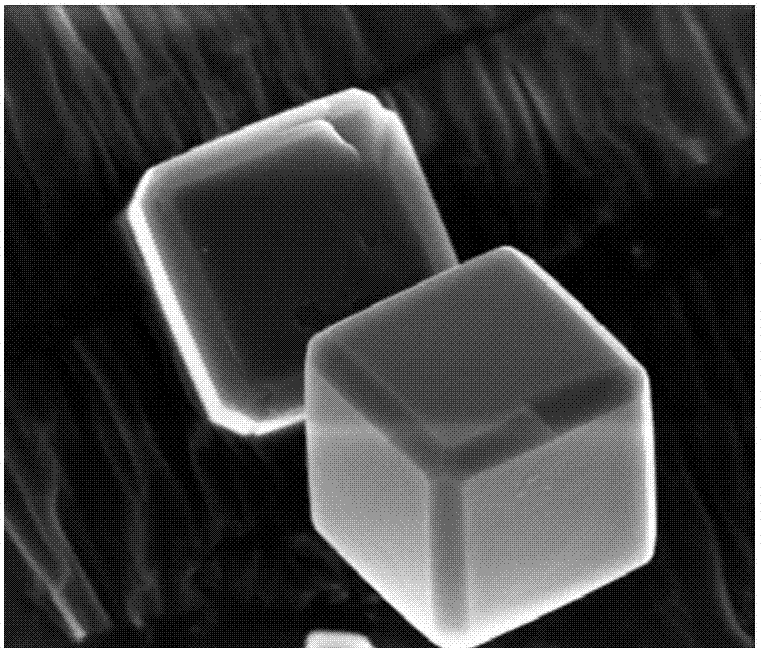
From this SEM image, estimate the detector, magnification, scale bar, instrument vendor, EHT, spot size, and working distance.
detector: TLD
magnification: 30.844 K X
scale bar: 3000 nm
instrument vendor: FEI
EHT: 5 kV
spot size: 3.5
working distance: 4.7 mm